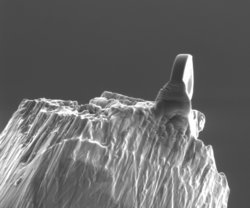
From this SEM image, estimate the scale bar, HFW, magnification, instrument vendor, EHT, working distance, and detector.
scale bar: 10000 nm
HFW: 23 µm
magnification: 6.5 K X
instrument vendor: FEI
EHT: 5 kV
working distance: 5 mm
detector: ETD